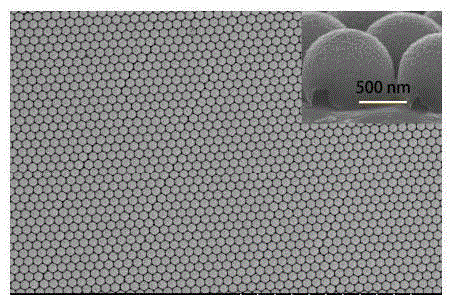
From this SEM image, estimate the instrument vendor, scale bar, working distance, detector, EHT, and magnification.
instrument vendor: Hitachi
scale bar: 20000 nm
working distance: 9.7 mm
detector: SE(M)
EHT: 15 kV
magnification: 2.5 K X